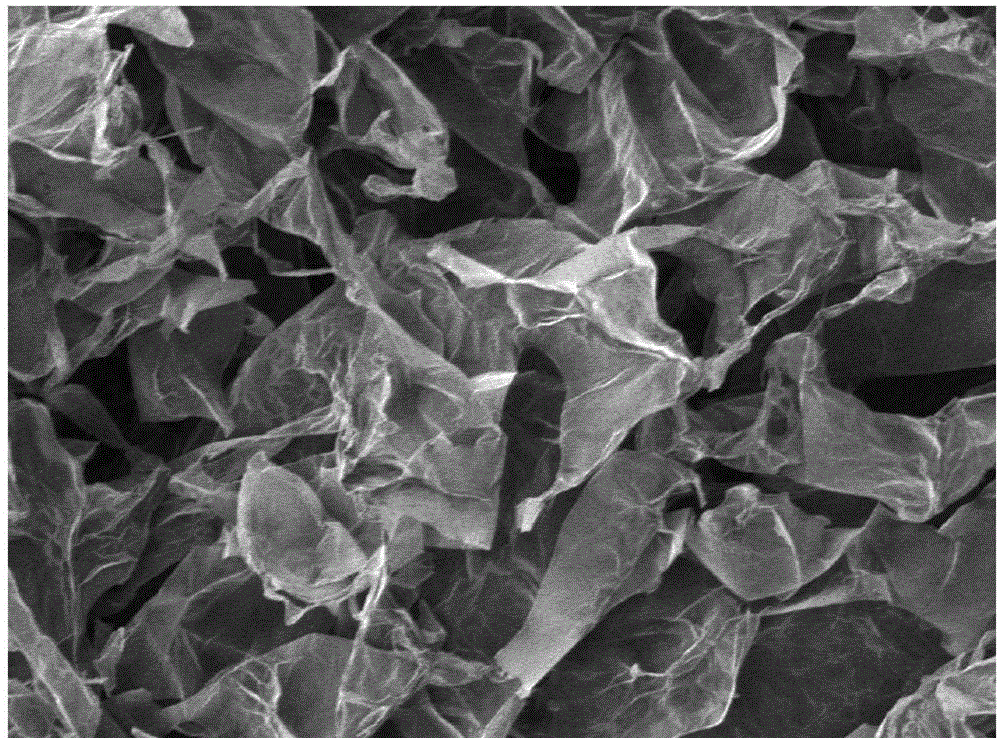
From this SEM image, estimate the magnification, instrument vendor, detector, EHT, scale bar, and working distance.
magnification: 0.5 K X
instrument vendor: JEOL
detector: SEI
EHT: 5 kV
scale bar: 10000 nm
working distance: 8 mm